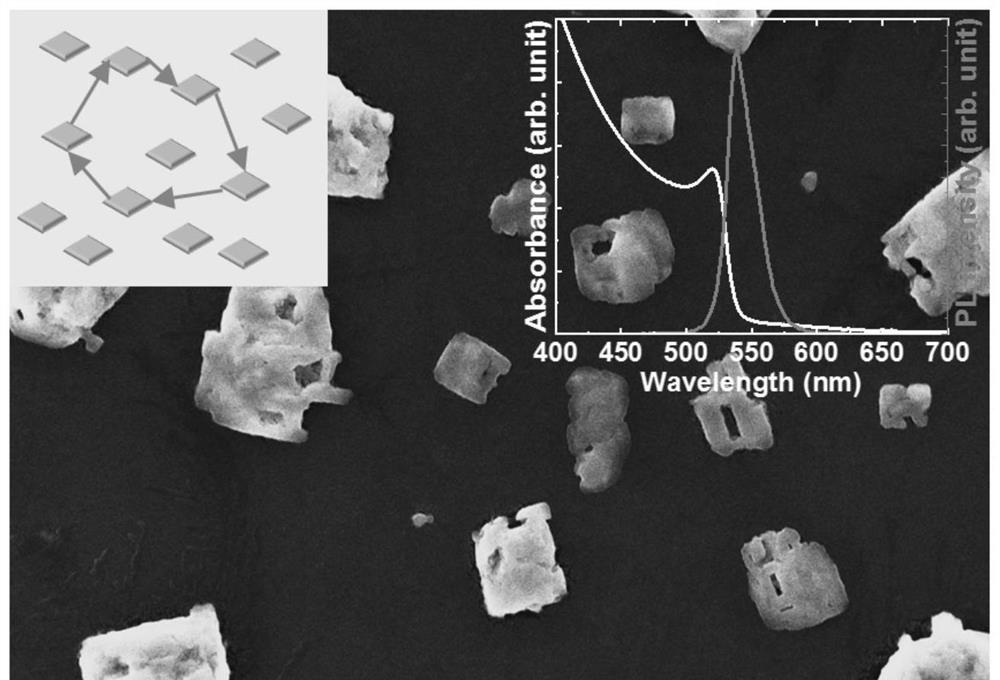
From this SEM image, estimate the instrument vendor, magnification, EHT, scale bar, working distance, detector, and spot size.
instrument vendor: JEOL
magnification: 2.7 K X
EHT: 15 kV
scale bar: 5000 nm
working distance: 10 mm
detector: SEI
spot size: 50